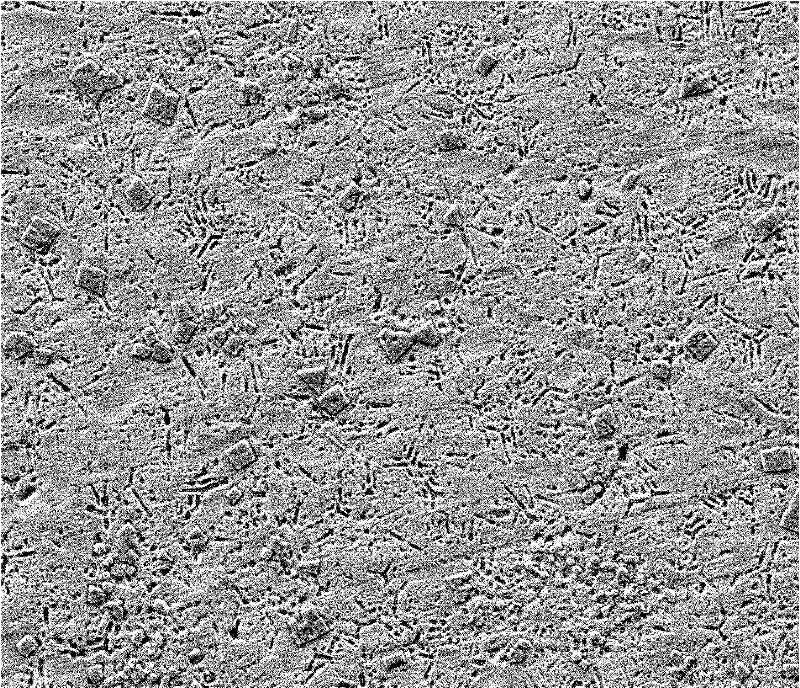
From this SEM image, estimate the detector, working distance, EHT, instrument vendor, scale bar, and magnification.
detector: SE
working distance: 10.8 mm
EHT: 20 kV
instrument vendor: FEI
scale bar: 100000 nm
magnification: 1 K X